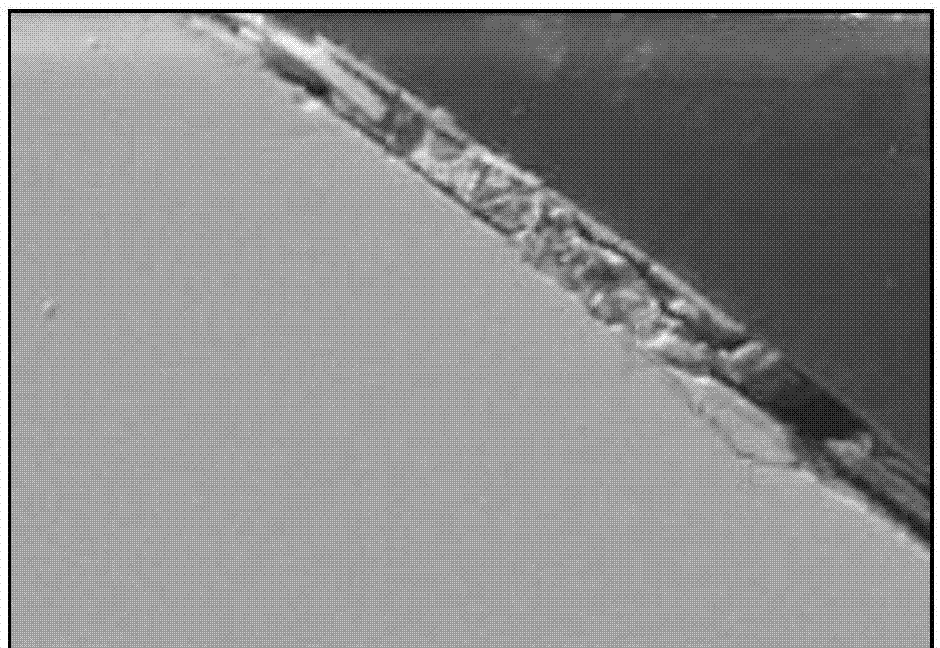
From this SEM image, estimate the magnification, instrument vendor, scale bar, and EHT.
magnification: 0.2 K X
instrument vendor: Hitachi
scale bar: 50000 nm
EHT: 22 kV